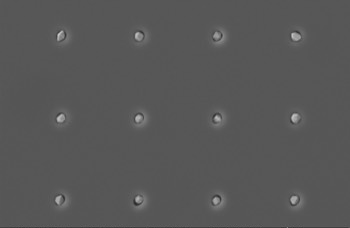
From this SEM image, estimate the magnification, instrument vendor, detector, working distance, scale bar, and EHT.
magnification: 13.51 K X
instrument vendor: Zeiss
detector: SE2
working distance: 7 mm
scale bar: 1000 nm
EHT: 5 kV